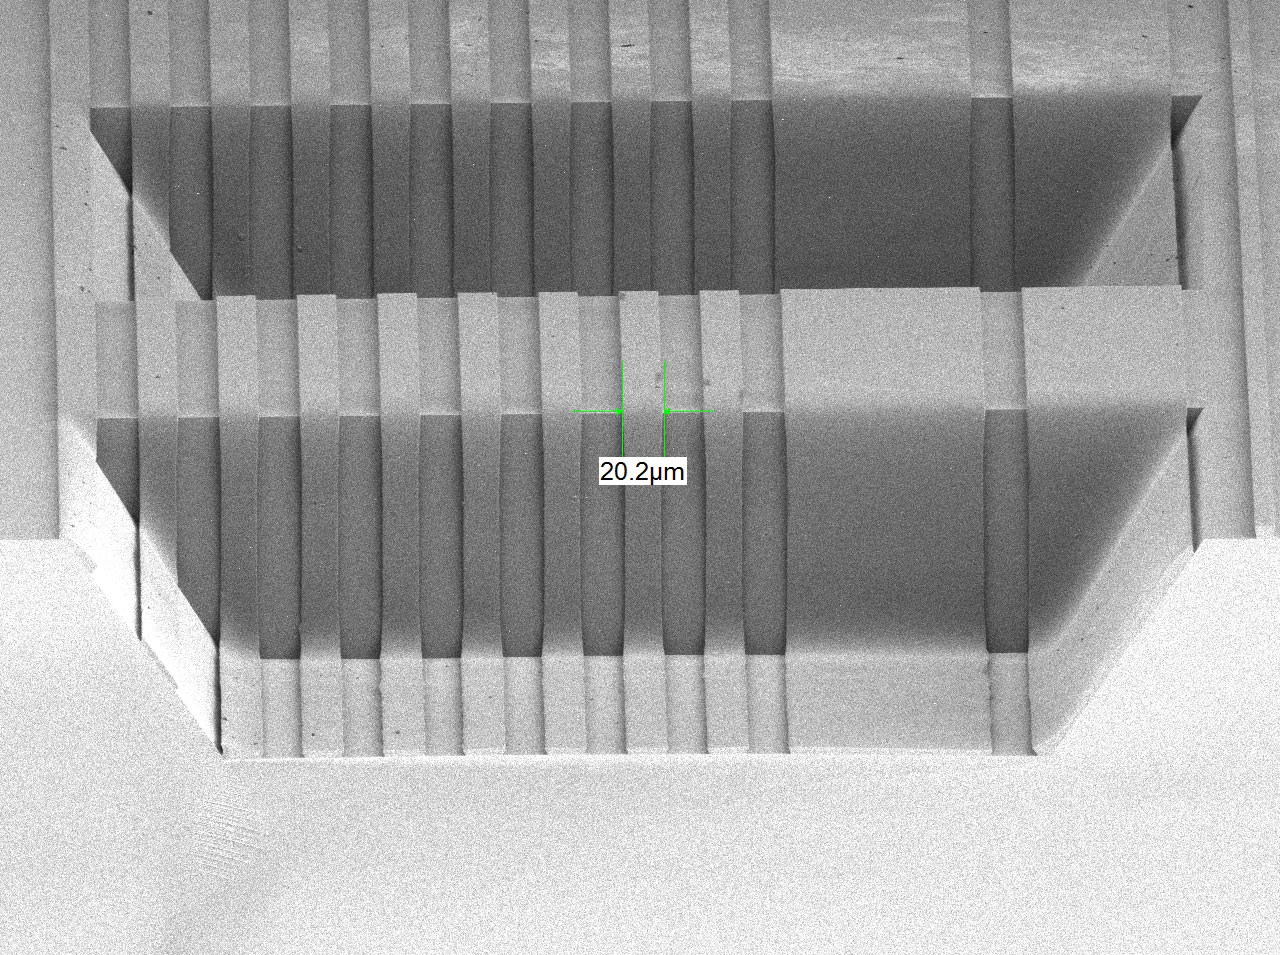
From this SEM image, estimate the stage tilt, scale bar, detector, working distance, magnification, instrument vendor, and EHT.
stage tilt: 6.6°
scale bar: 100000 nm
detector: SEI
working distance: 8 mm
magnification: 0.19 K X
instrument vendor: JEOL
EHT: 2 kV